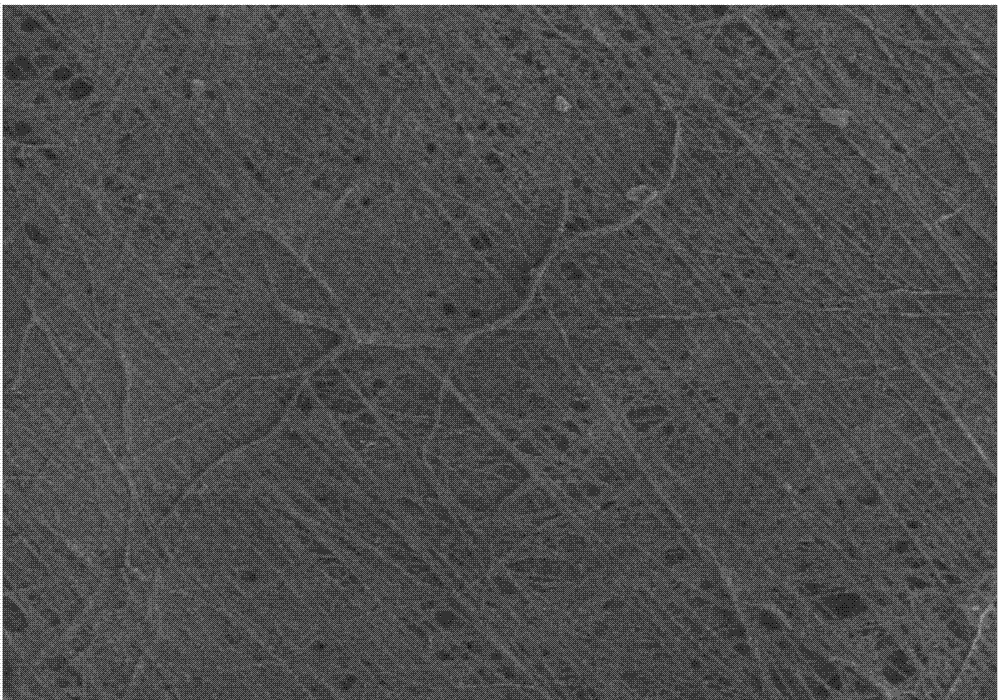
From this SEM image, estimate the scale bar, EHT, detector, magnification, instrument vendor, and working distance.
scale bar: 30000 nm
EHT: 5 kV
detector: HE-SE2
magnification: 0.5 K X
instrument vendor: Zeiss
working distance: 6 mm